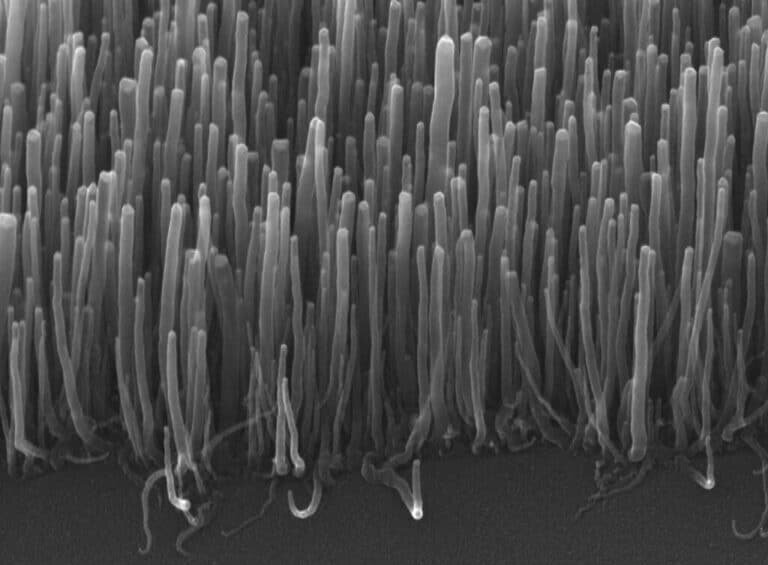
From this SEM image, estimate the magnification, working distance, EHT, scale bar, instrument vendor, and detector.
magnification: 22 K X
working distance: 15.3 mm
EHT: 30 kV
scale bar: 1000 nm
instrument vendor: JEOL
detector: SEI